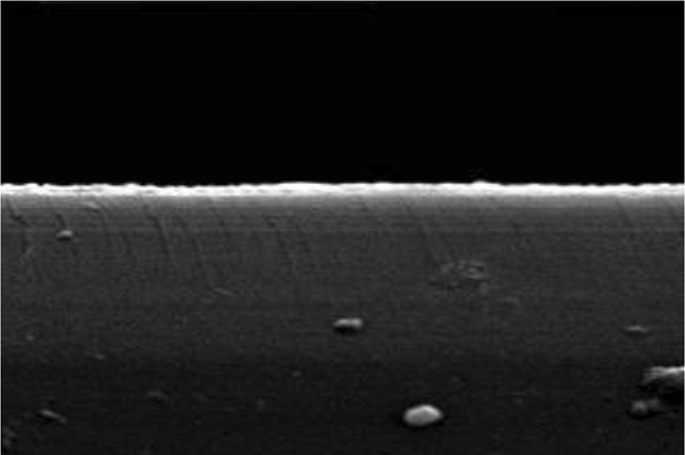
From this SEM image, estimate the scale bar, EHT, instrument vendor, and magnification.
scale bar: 3000 nm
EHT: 20 kV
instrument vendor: JEOL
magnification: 10 K X